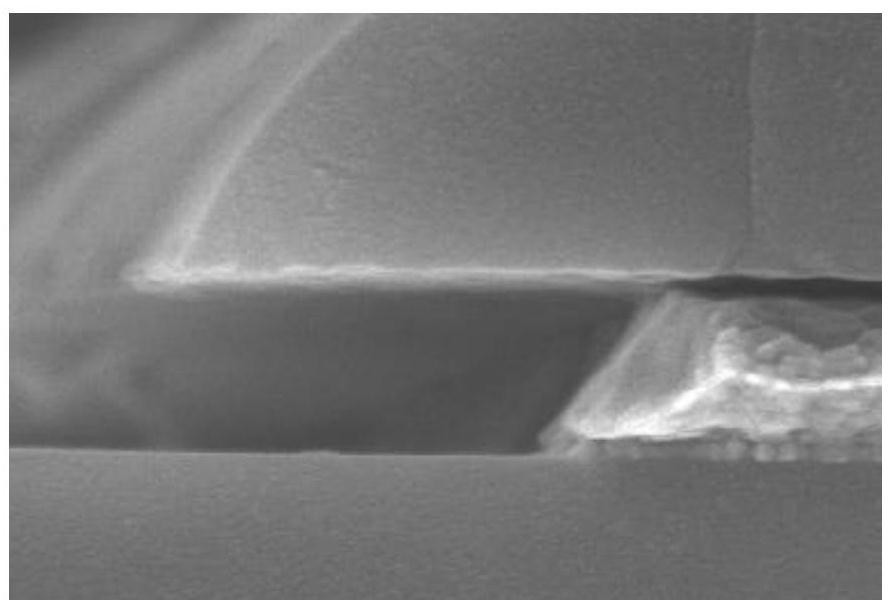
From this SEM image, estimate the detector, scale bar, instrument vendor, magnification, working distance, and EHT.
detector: SE(UL)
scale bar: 500 nm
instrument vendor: Hitachi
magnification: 80 K X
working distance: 10.4 mm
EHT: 5 kV